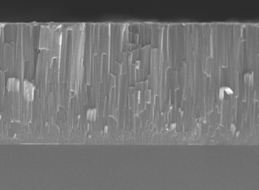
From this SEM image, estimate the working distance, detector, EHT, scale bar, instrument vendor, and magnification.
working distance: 4.1 mm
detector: SEI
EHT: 15 kV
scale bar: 1000 nm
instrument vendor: JEOL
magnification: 25 K X